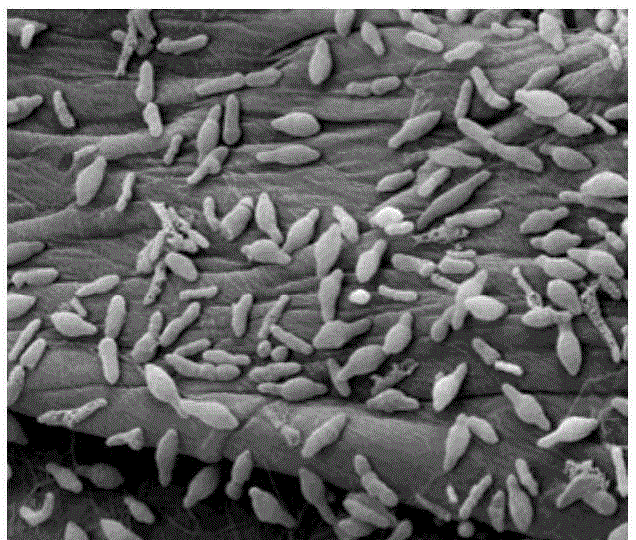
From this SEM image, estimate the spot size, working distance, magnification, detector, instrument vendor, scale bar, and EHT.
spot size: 3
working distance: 11.2 mm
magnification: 5 K X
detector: ETD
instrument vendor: FEI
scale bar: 10000 nm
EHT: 12.5 kV